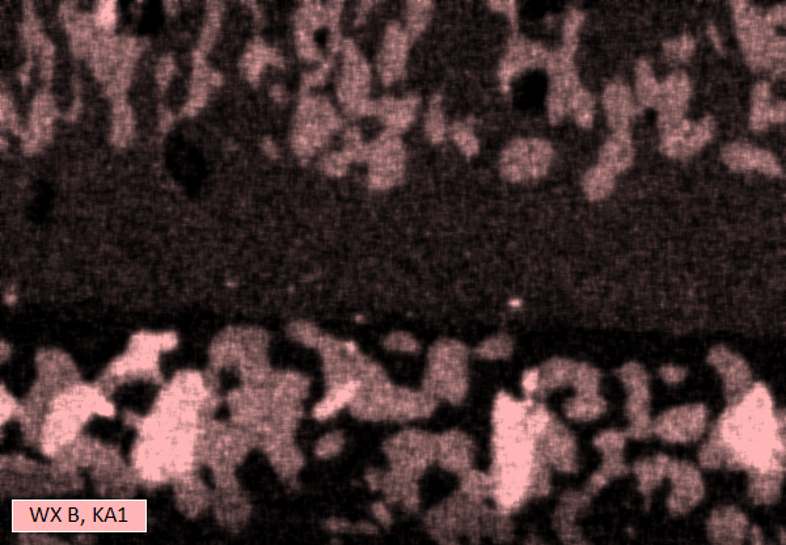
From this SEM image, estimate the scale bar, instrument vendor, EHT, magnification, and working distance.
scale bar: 5000 nm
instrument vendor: other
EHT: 5 kV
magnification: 4.5 K X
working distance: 15 mm